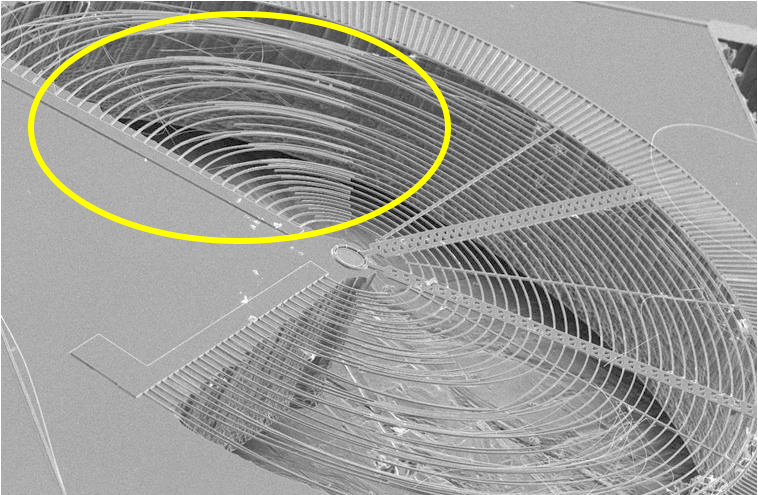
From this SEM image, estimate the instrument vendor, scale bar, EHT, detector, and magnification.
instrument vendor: JEOL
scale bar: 200000 nm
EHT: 20 kV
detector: SEI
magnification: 0.065 K X